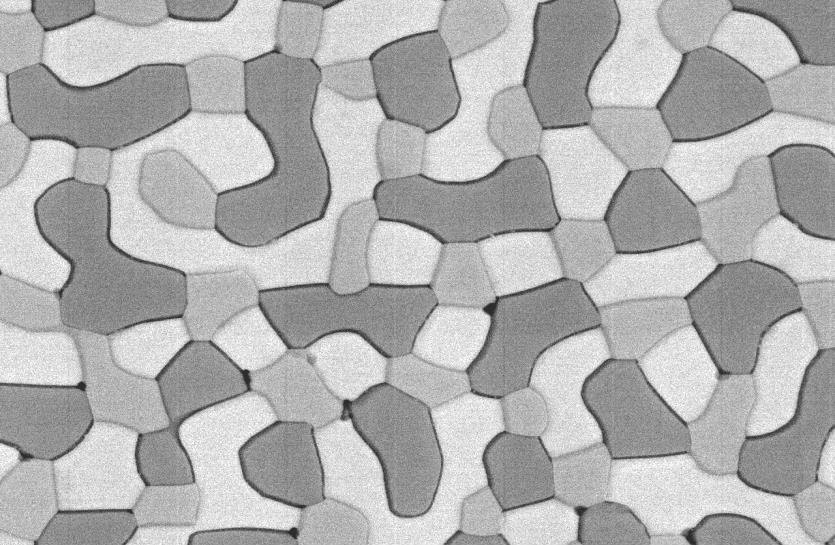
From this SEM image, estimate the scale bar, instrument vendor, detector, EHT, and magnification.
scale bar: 1000 nm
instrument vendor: JEOL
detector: BEI COMPO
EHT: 10 kV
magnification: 6 K X